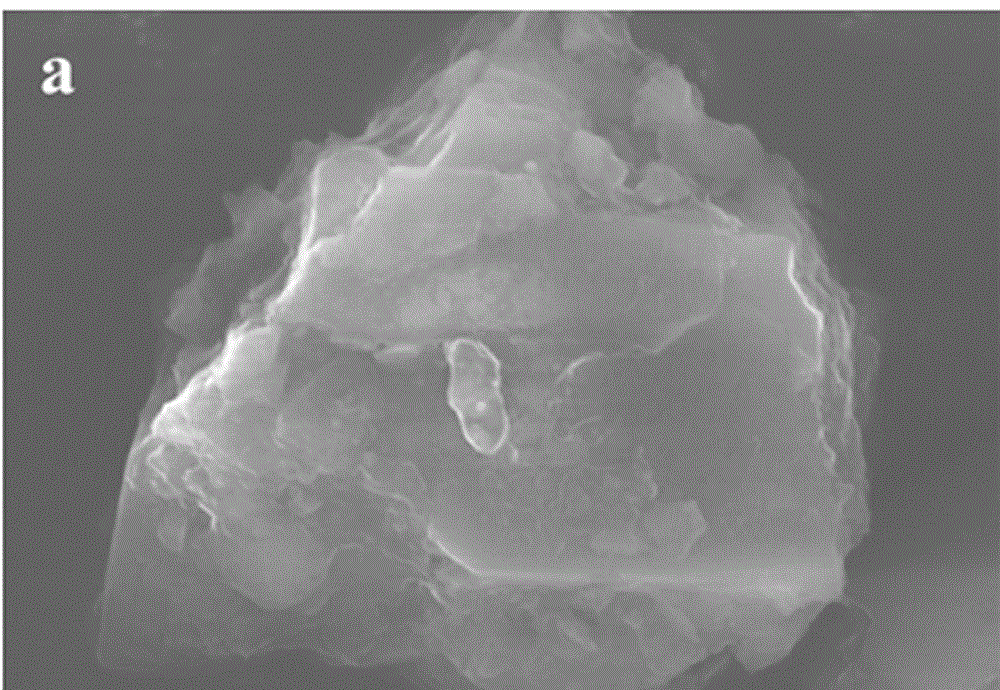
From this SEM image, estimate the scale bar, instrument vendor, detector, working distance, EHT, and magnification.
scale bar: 3000 nm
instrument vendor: Hitachi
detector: SE(UL)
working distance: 8.4 mm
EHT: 15 kV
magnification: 15 K X